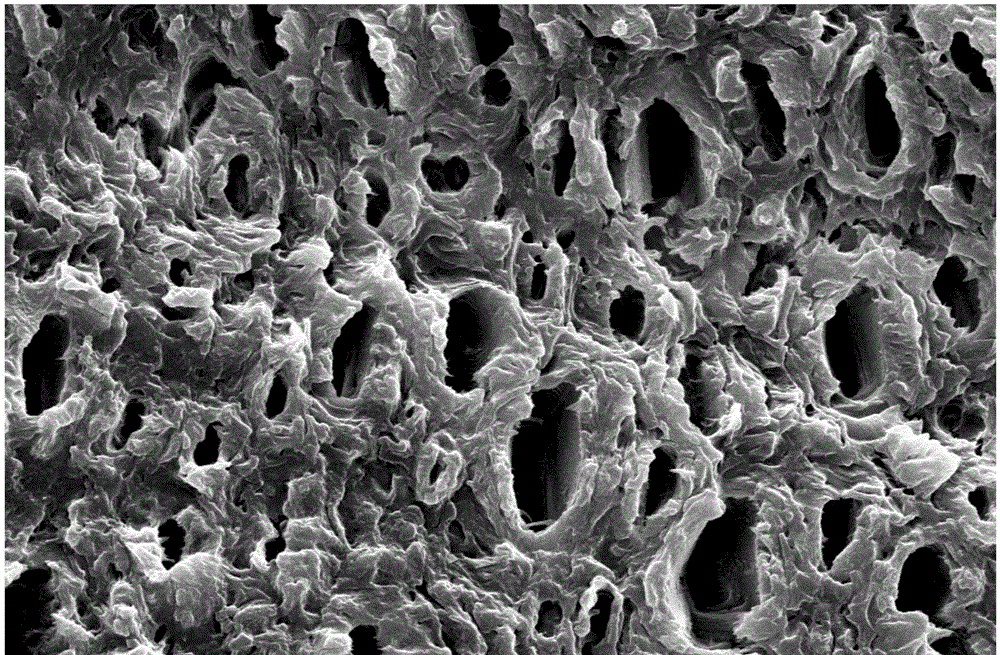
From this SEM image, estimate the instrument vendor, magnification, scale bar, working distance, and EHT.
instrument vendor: Zeiss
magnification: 5 K X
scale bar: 2000 nm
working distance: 12 mm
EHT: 20 kV